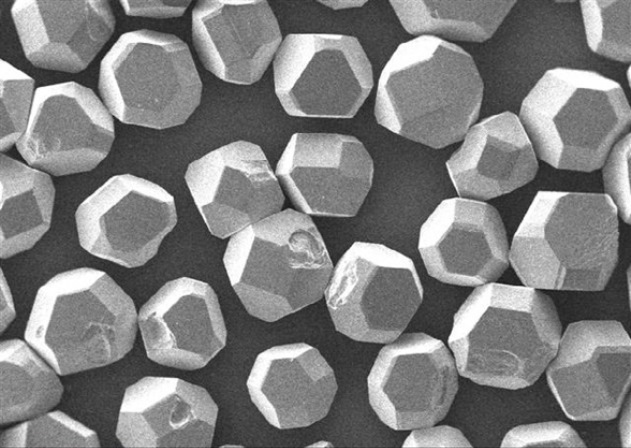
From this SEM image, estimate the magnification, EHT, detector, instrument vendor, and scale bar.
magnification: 0.05 K X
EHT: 20 kV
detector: SED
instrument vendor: other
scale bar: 500000 nm